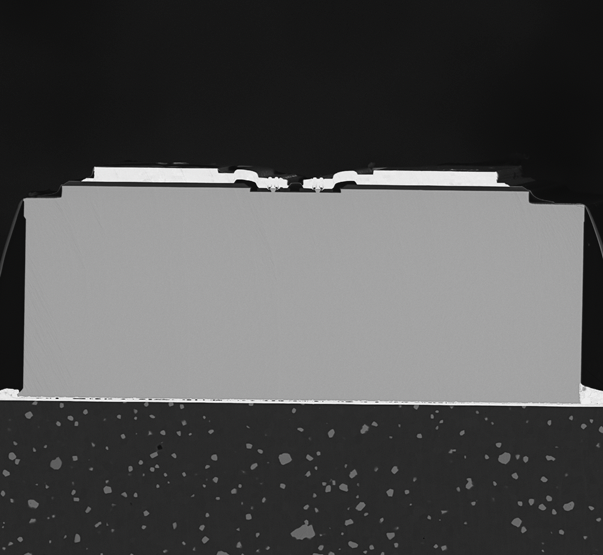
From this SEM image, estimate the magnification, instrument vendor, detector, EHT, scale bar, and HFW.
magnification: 1 K X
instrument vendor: other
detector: BSD Full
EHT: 15 kV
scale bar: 80000 nm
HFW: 269 µm